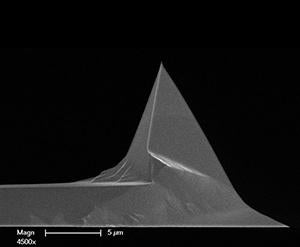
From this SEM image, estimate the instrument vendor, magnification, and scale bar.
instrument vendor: FEI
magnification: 4.5 K X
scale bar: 5000 nm